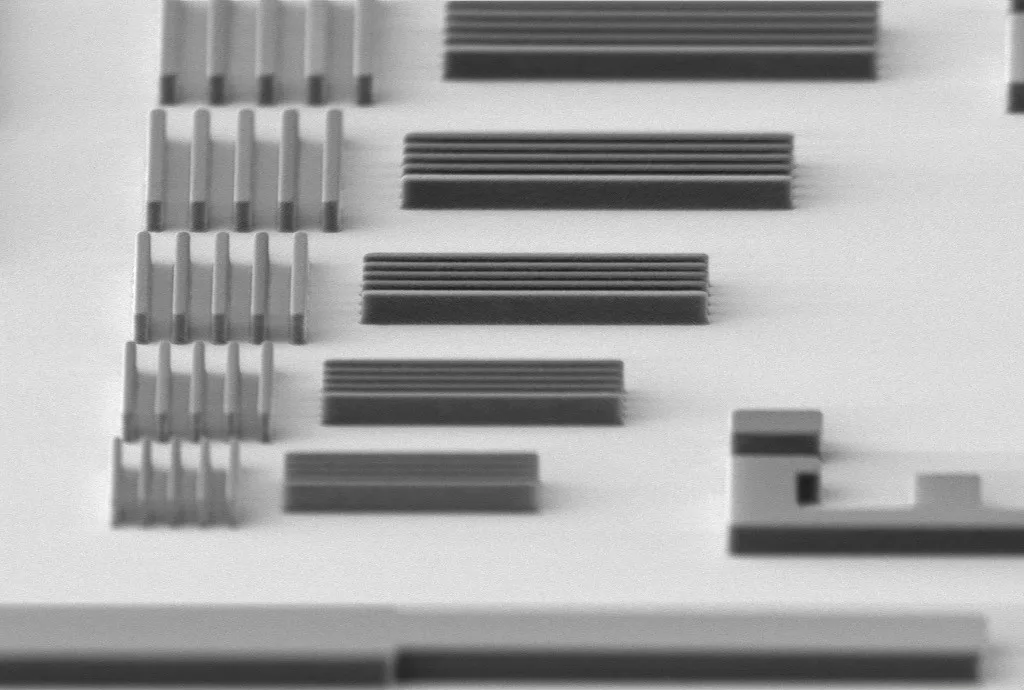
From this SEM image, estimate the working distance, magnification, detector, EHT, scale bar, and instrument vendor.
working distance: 10.8 mm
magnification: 13.4 K X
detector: SE2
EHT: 3 kV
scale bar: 2000 nm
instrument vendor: Zeiss